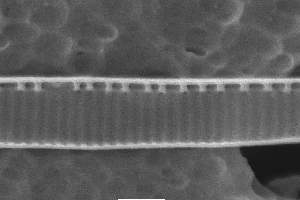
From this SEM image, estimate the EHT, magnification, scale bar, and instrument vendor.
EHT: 10 kV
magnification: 20 K X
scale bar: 1000 nm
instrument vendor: JEOL